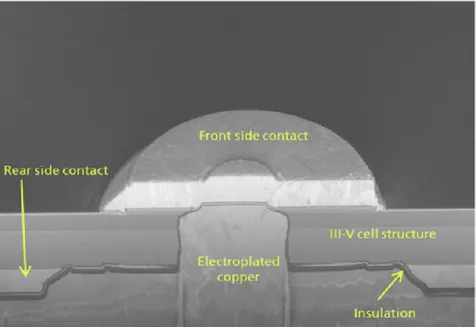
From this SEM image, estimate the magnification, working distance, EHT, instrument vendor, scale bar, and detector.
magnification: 3.16 K X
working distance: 5 mm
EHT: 2 kV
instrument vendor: Zeiss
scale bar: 2000 nm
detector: InLens